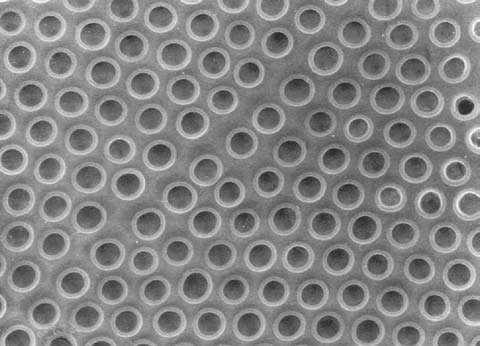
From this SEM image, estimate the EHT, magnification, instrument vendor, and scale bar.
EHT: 10 kV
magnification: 10 K X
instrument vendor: JEOL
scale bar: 3000 nm